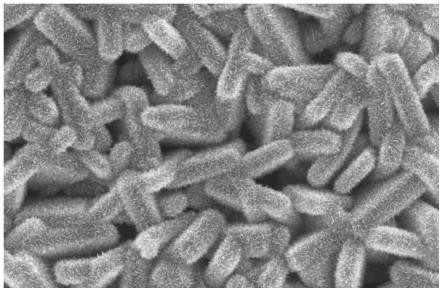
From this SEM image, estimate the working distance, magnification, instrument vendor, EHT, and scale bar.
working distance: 9.4 mm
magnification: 20 K X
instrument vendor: Hitachi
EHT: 10 kV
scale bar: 2000 nm